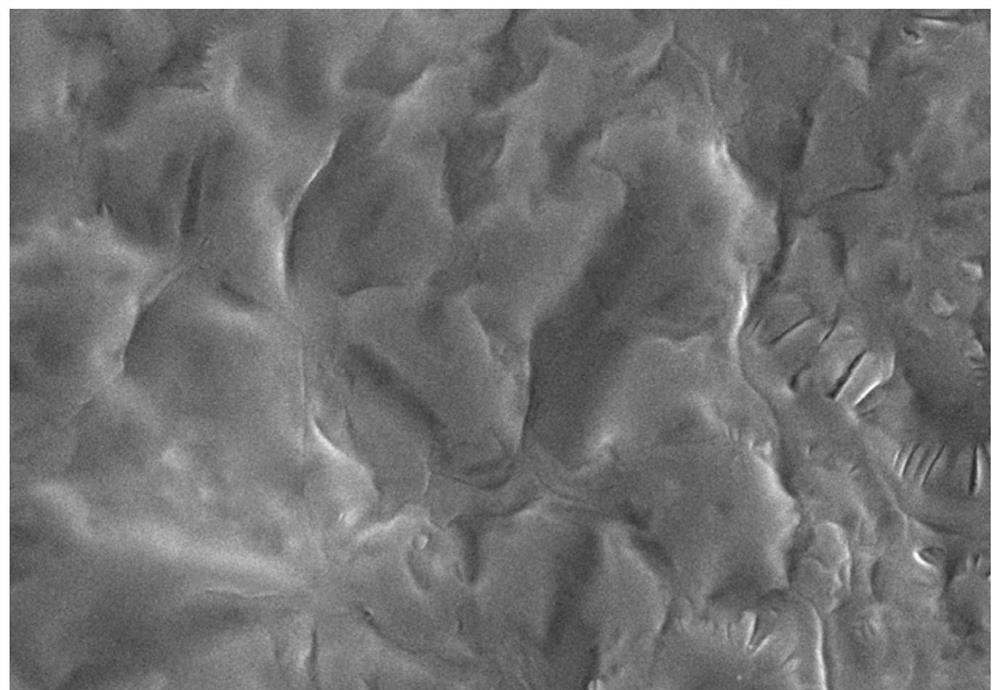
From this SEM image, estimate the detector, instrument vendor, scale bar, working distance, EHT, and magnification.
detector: SE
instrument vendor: Hitachi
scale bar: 10000 nm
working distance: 5.5 mm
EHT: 10 kV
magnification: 5 K X